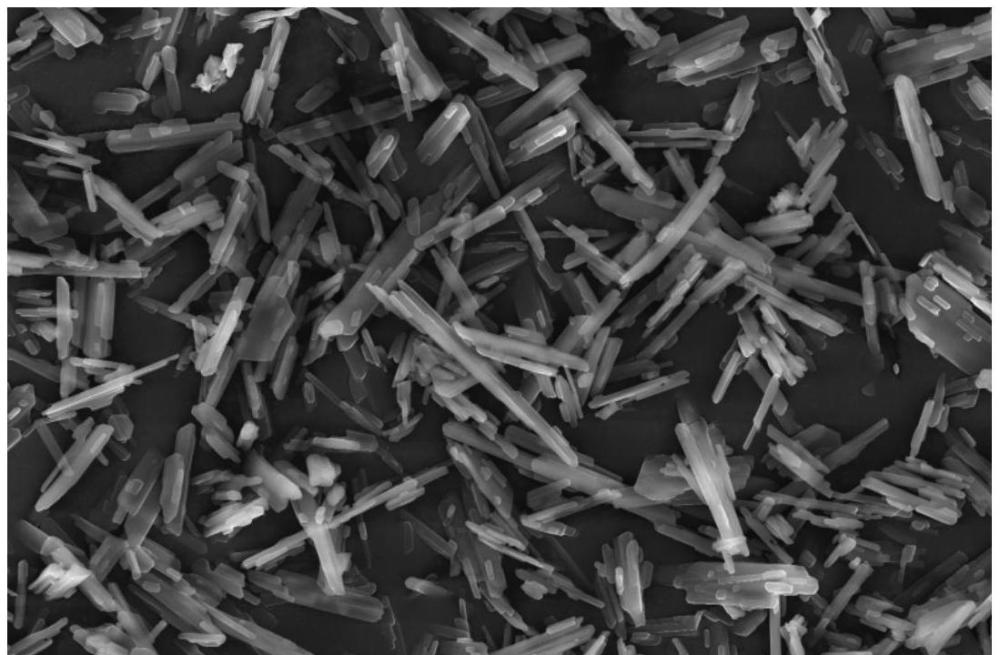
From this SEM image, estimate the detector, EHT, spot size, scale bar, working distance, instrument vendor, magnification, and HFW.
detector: ETD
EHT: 30 kV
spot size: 10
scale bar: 30000 nm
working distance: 10.2 mm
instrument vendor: Thermo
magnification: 2 K X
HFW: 104 µm